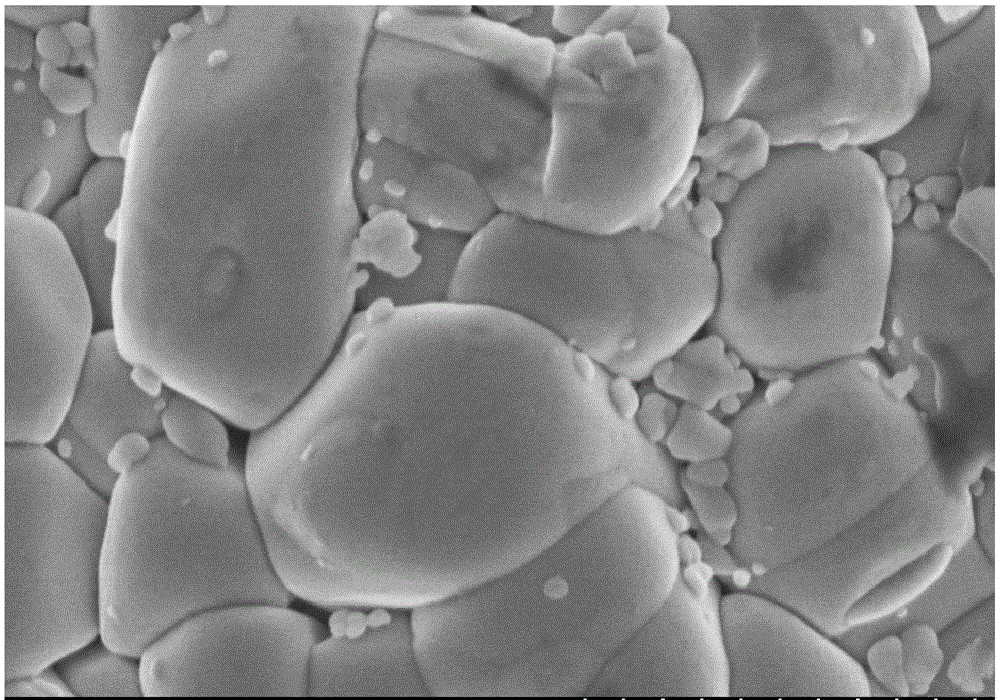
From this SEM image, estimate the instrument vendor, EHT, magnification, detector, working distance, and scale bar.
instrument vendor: Hitachi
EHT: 3 kV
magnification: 50 K X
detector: SE(M)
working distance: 8.1 mm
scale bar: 1000 nm